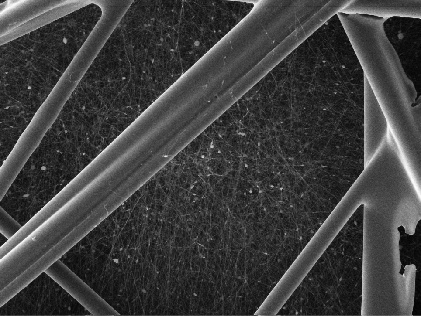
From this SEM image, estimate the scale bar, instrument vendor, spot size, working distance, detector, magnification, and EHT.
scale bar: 20000 nm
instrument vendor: other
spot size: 1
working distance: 7.51 mm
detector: SEI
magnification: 0.5 K X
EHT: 20 kV